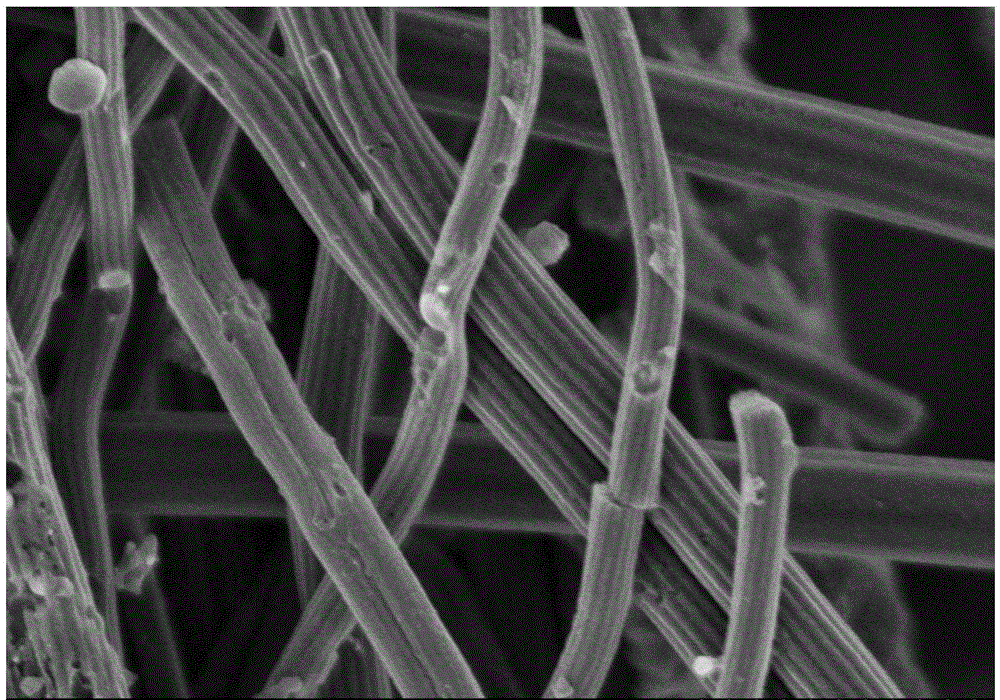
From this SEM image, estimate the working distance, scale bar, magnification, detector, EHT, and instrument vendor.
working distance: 5.2 mm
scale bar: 1000 nm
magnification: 35 K X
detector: SE(M)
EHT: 2 kV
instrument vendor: Hitachi